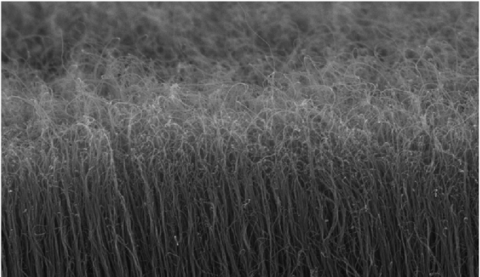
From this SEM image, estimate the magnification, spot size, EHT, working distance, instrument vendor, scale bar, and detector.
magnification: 20 K X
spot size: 2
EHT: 5 kV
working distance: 6.3 mm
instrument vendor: FEI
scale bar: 1000 nm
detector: TLD